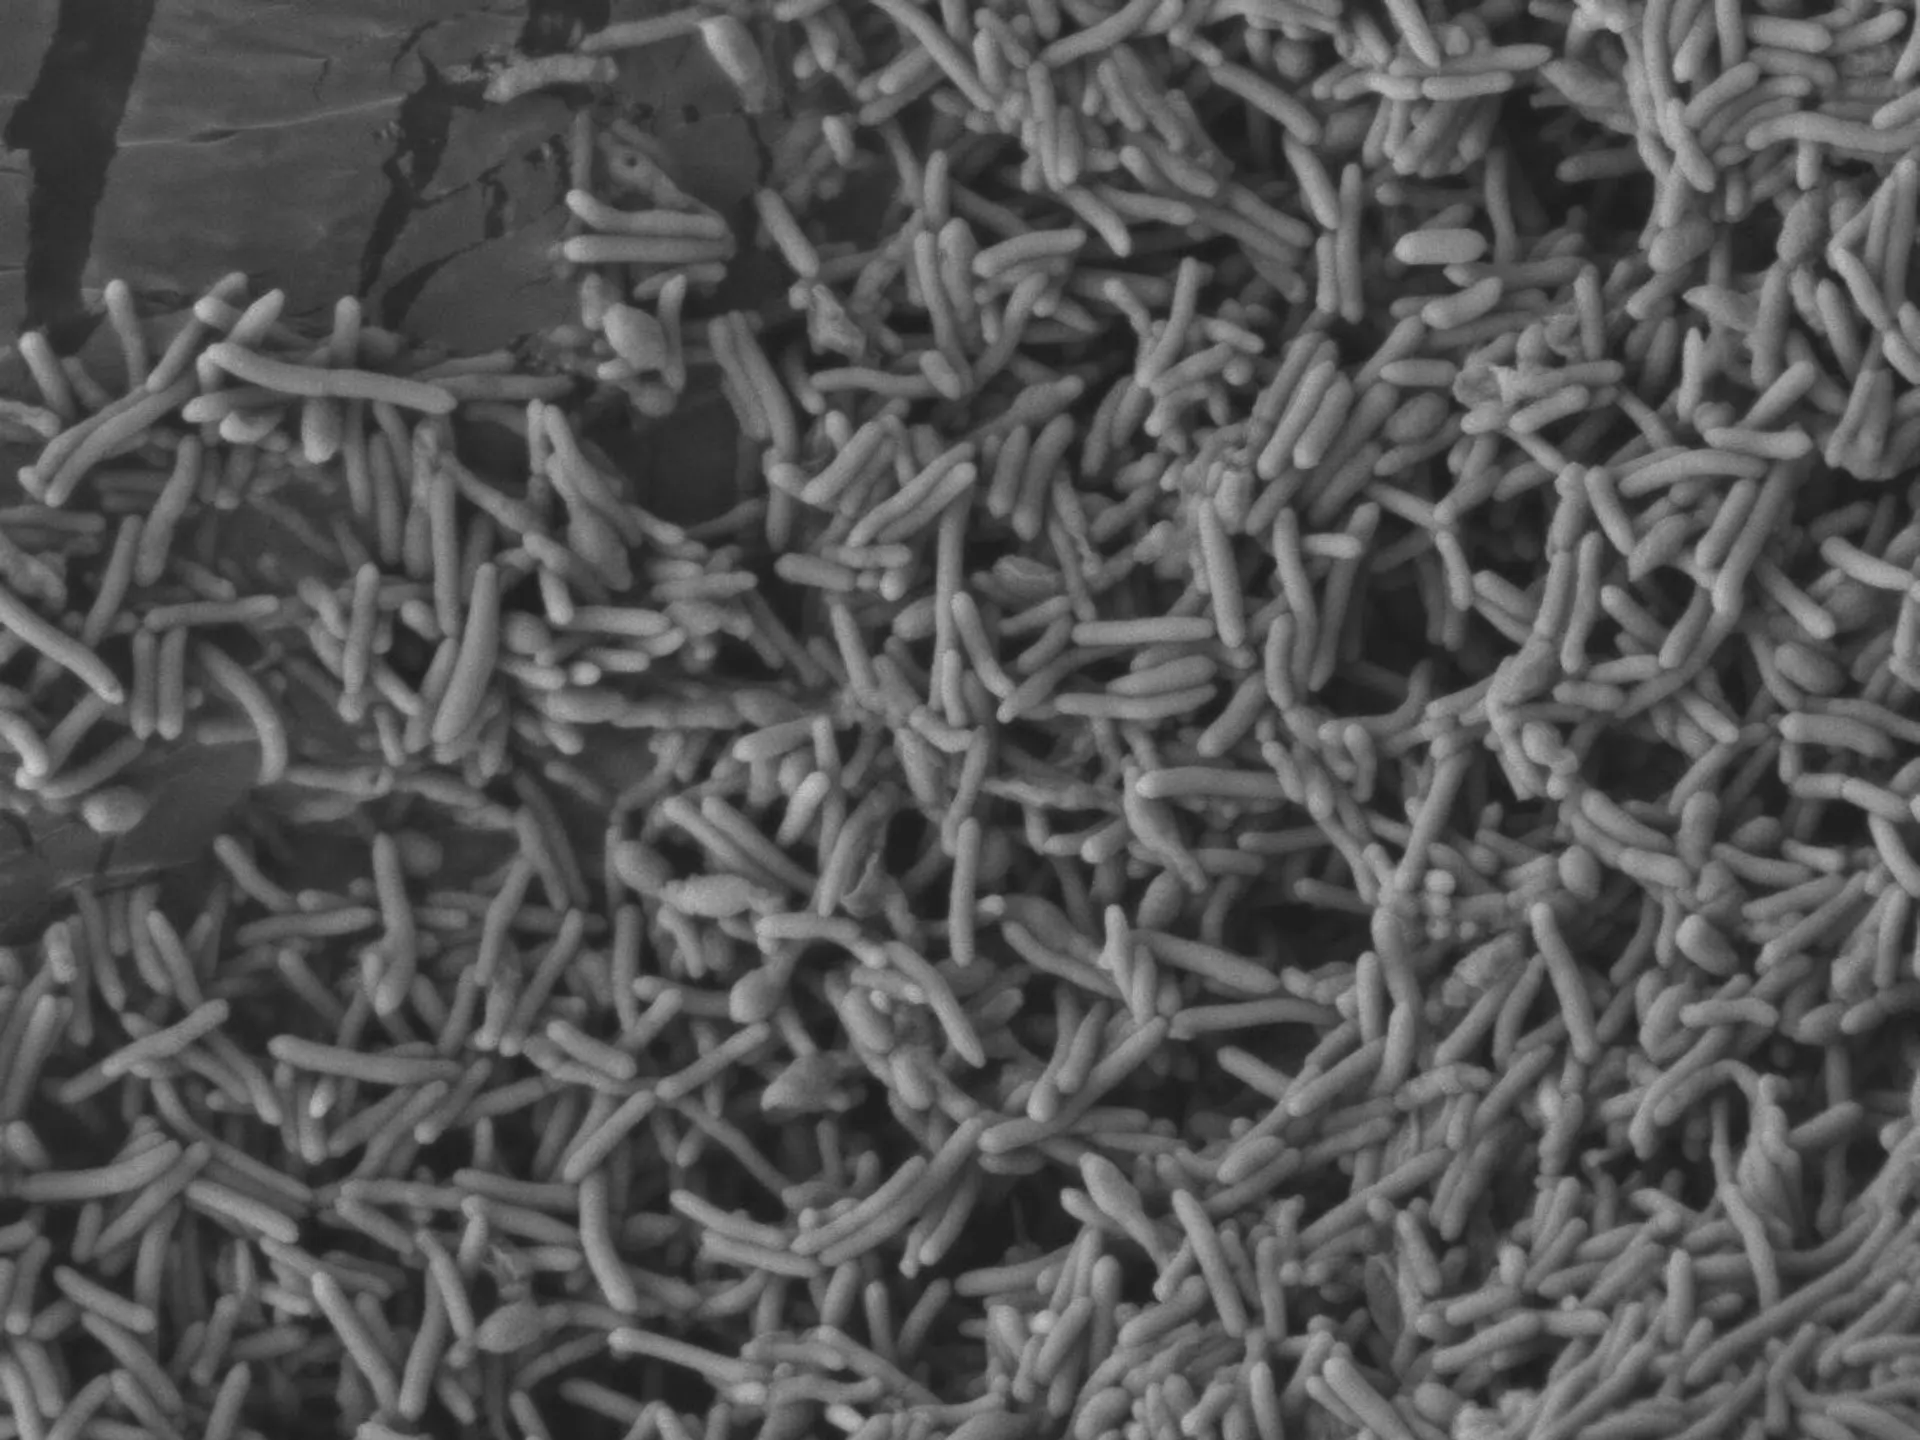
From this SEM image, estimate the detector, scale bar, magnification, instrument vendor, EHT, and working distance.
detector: LEI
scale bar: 1000 nm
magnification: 3 K X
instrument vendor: JEOL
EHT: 5 kV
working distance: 8 mm